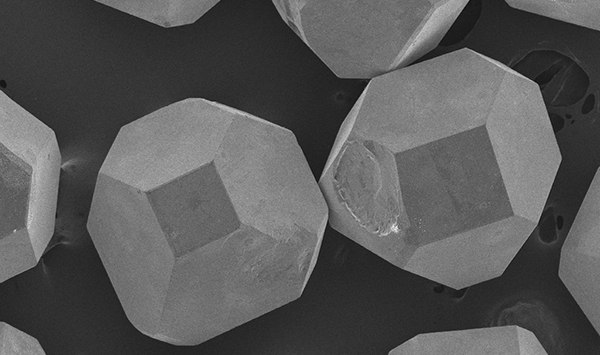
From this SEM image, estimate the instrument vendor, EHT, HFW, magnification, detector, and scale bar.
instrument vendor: JEOL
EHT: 5 kV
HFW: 1490 µm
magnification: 0.18 K X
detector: SED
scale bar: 300000 nm